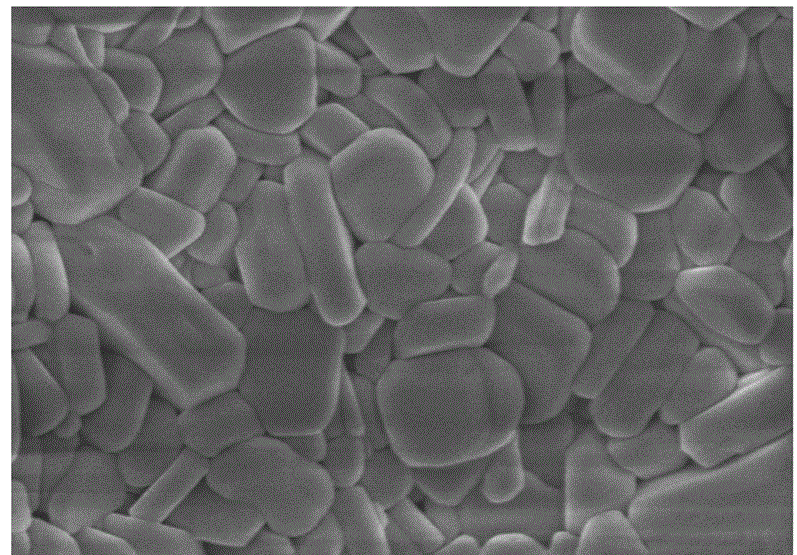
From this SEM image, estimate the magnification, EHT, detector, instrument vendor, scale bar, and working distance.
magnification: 35 K X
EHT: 3 kV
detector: SE(M)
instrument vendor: Hitachi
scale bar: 1000 nm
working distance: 8.5 mm